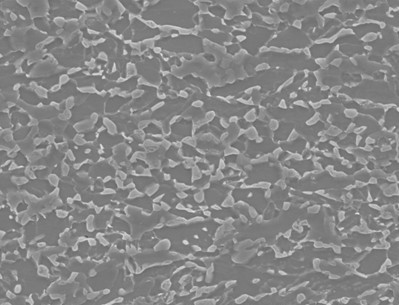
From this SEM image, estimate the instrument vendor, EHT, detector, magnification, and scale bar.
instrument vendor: JEOL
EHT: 25 kV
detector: SEI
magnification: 1.5 K X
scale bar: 10000 nm